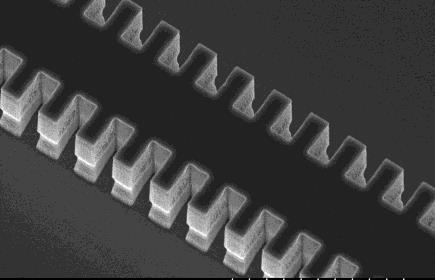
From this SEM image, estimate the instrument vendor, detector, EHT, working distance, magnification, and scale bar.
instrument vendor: Hitachi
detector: SE(U)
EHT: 10 kV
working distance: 11.8 mm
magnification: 5 K X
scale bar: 10000 nm